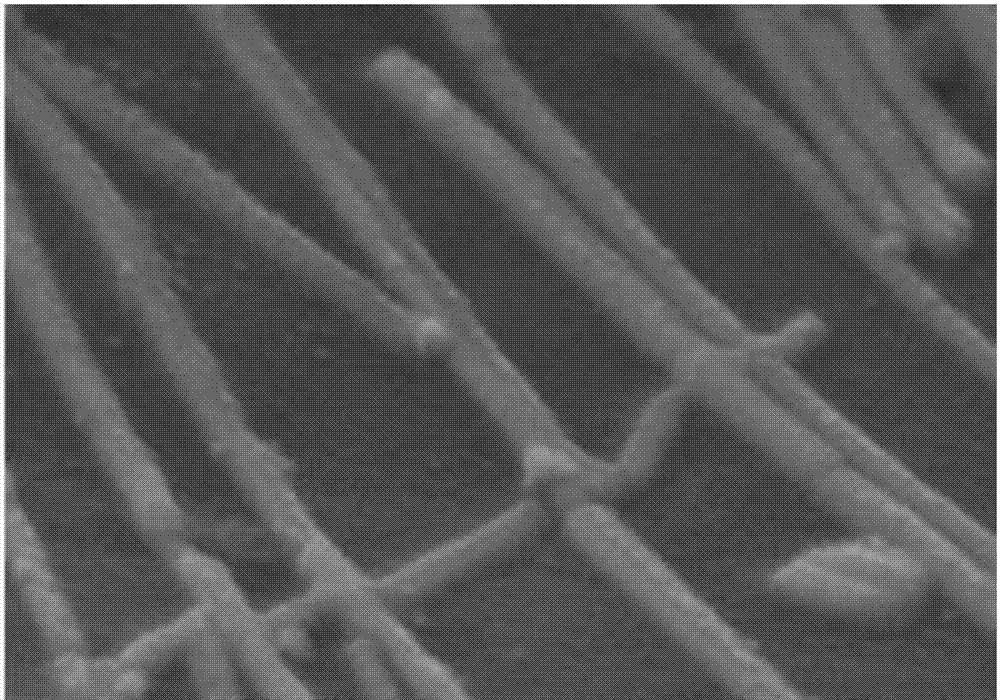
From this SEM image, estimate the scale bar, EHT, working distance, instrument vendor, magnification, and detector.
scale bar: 500 nm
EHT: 5 kV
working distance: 9.9 mm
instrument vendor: Hitachi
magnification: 100 K X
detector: SE(M)